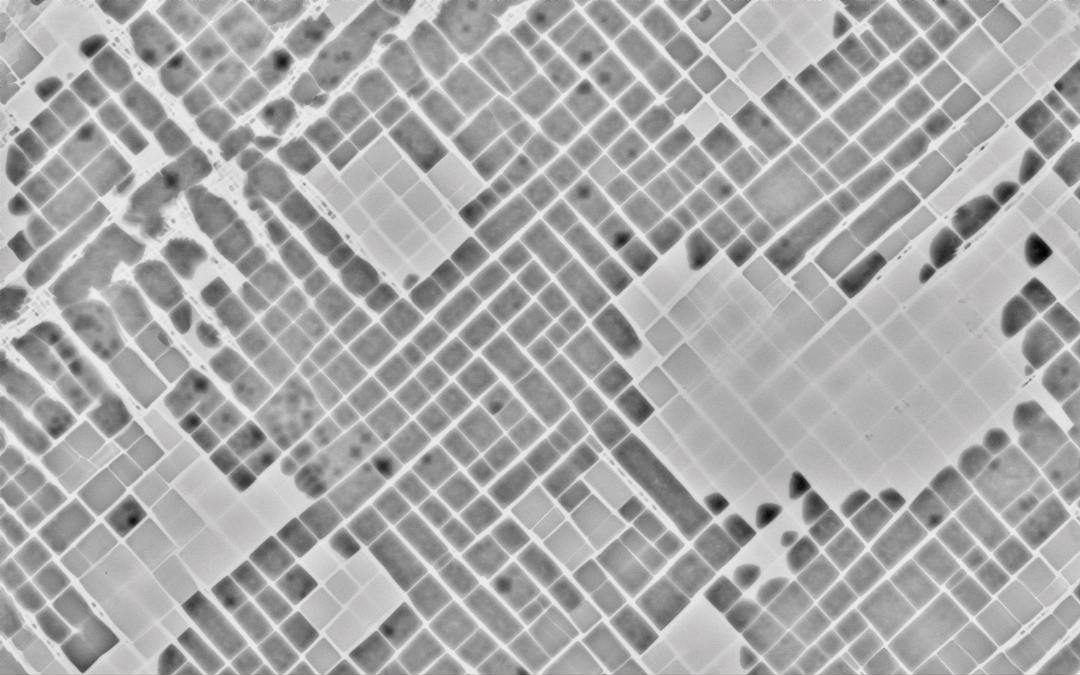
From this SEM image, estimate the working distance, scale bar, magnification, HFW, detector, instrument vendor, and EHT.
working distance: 9.191 mm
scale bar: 3000 nm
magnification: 50 K X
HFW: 10.4 µm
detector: BSD Full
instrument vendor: Thermo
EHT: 15 kV